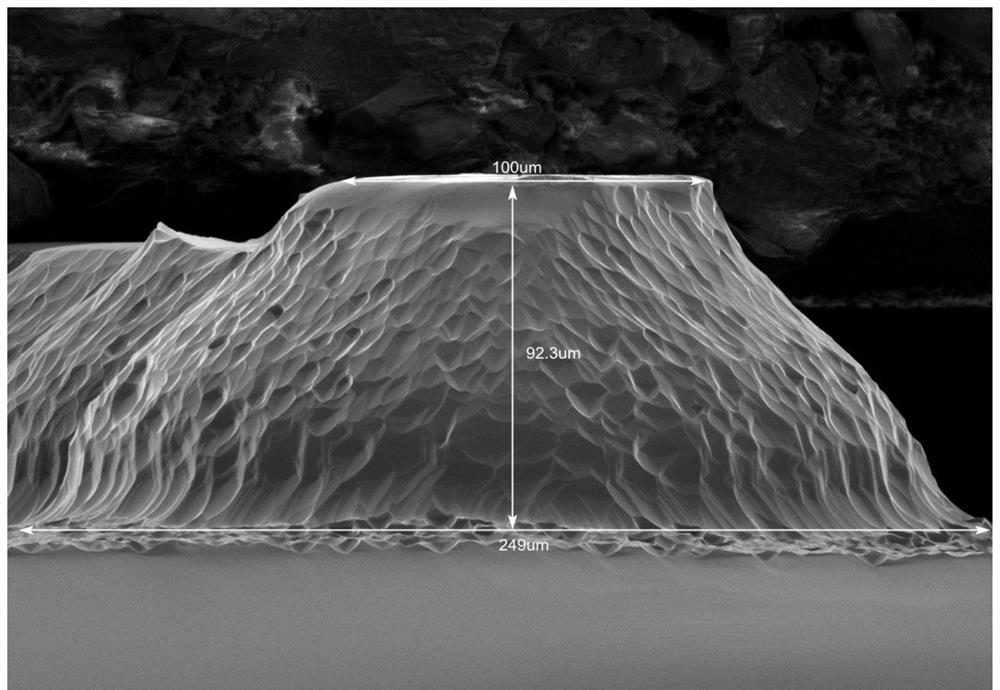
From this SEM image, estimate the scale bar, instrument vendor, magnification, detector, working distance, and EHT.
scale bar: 100000 nm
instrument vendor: Hitachi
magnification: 0.5 K X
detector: LM(UL)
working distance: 8.7 mm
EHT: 3 kV